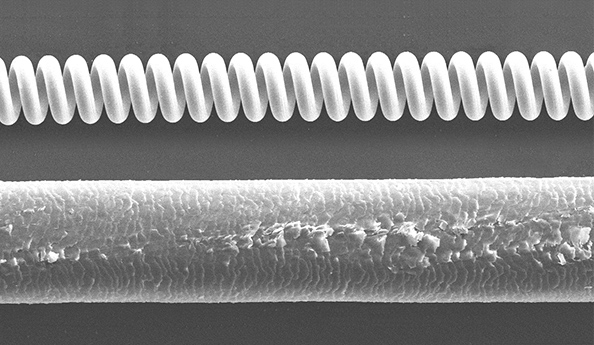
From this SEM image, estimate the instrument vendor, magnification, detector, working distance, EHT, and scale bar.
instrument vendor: JEOL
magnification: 0.2 K X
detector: SEI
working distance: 11 mm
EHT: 15 kV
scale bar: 100000 nm